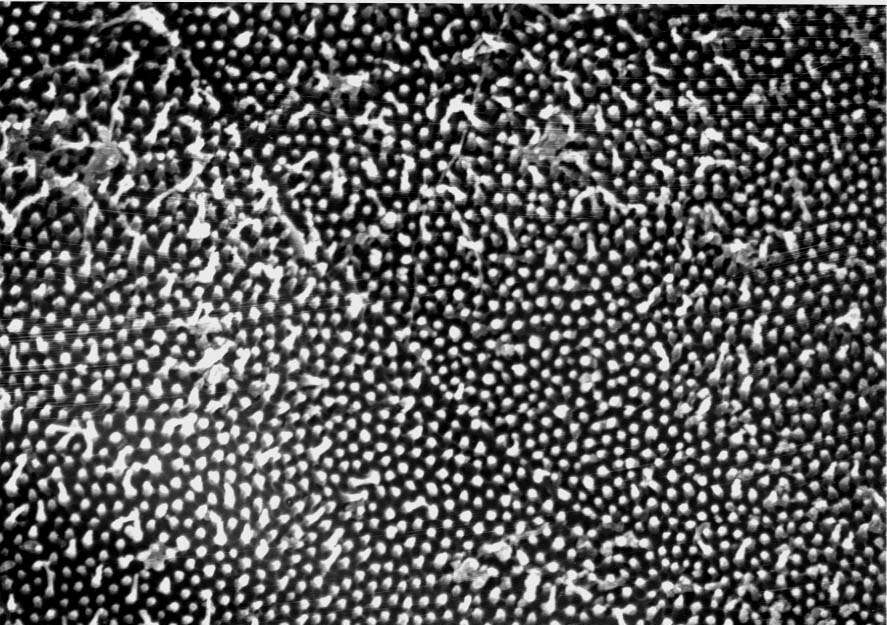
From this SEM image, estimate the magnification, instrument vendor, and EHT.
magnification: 5.7 K X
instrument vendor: JEOL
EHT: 20 kV